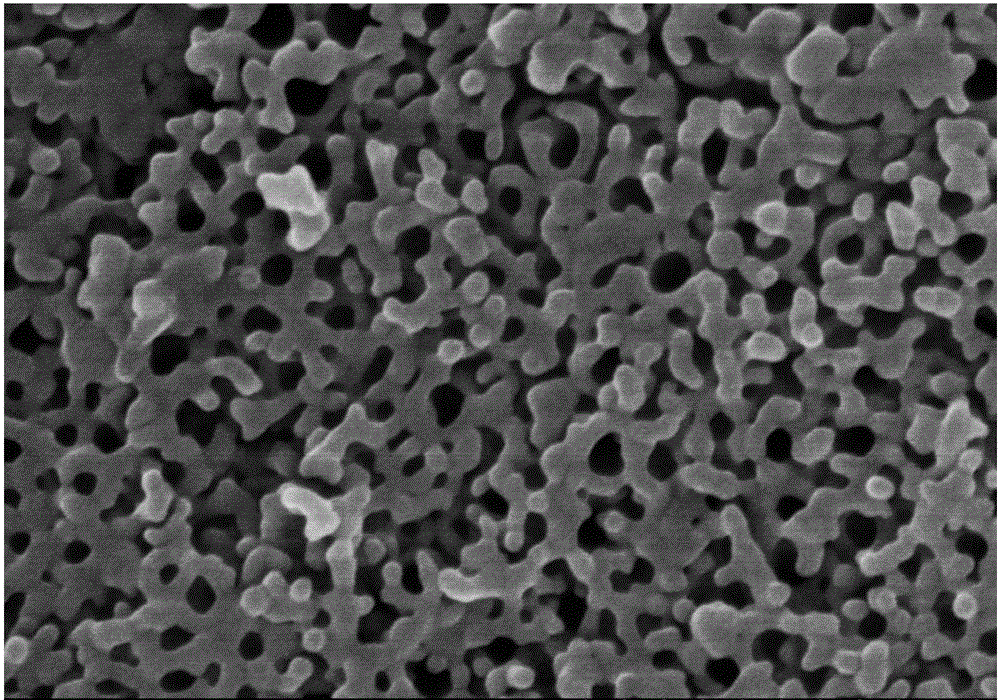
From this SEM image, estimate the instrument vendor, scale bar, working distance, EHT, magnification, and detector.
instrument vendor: Hitachi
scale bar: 500 nm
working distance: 8.3 mm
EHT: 10 kV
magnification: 100 K X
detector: SE(M,LA0)